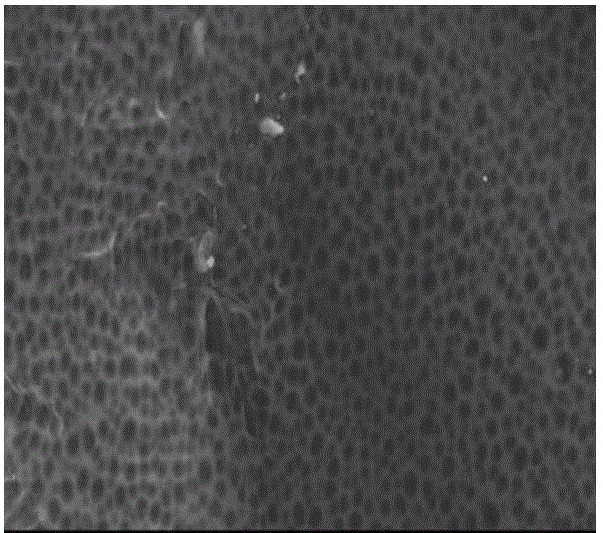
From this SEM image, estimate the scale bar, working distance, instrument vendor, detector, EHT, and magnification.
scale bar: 50000 nm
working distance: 9 mm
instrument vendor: JEOL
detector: SEI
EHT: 30 kV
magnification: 0.33 K X